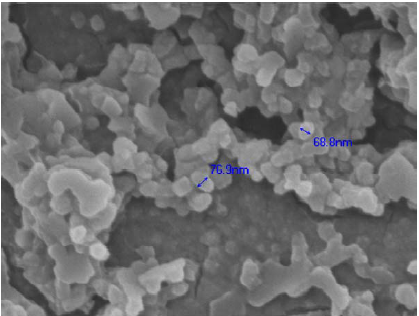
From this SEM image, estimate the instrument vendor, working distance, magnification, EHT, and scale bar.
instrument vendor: JEOL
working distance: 11.7 mm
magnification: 50 K X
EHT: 5 kV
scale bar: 100 nm